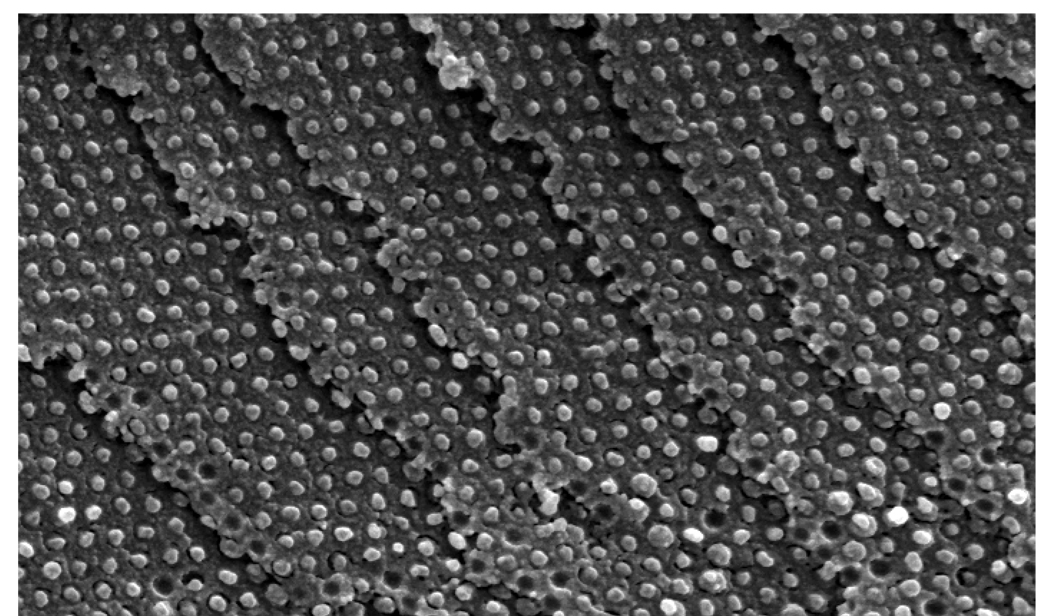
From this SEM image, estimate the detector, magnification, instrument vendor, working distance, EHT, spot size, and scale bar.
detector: SE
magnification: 15 K X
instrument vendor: FEI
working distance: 10 mm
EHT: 10 kV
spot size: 3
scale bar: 2000 nm